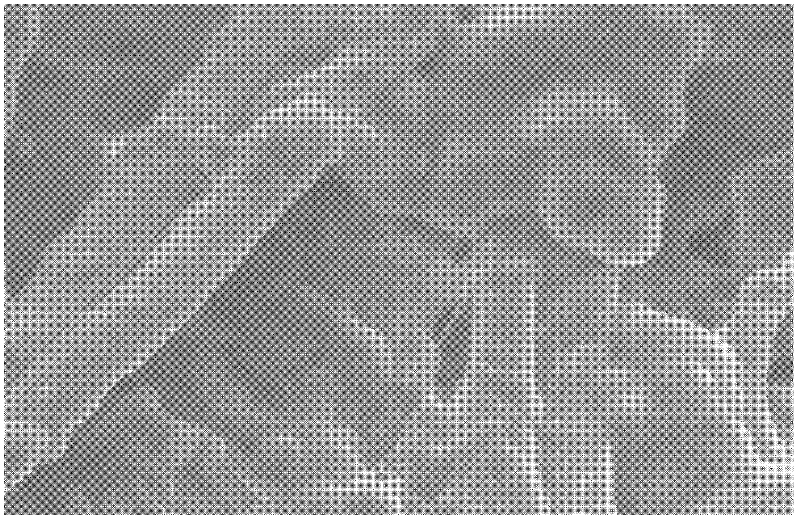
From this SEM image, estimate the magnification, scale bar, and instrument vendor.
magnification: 1.5 K X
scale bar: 10000 nm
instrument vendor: JEOL